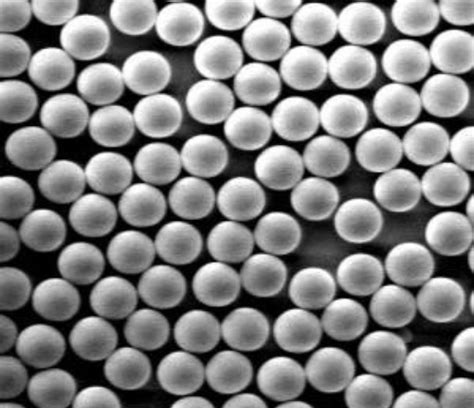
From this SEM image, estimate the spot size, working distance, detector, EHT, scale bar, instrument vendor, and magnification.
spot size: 3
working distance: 5 mm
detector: SE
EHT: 5 kV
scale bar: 2000 nm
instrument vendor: FEI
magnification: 10 K X